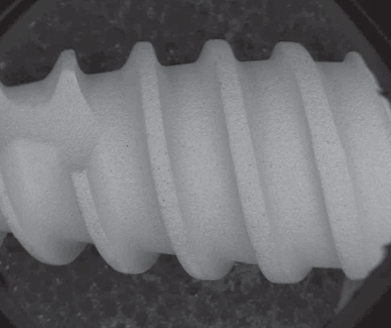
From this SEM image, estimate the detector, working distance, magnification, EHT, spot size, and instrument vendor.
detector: BSED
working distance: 10.3 mm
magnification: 0.05 K X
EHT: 20 kV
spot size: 4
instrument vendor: FEI